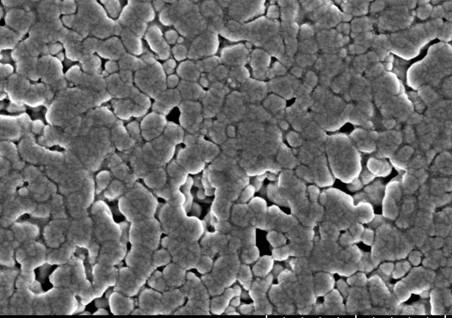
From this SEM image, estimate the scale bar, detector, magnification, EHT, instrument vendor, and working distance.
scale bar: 1000 nm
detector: SE(TUL)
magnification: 50 K X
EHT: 3 kV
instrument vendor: Hitachi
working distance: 8.1 mm